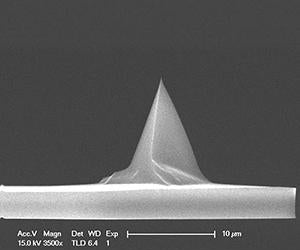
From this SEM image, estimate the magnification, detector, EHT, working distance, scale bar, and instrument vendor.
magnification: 3.5 K X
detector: TLD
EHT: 15 kV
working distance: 6.4 mm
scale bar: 10000 nm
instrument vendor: FEI